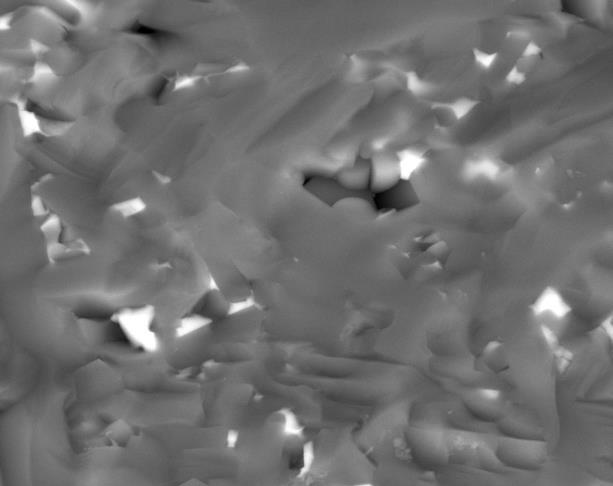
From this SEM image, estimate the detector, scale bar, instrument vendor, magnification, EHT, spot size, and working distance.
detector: BSED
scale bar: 10000 nm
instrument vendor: FEI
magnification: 5 K X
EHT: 30 kV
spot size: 4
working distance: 11.3 mm